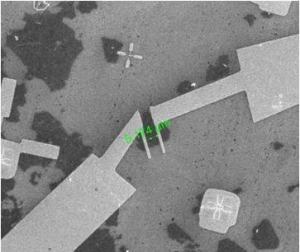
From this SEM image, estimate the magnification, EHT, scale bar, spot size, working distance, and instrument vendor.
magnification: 2.5 K X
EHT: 20 kV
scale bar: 50000 nm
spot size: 3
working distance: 8.6 mm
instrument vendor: FEI